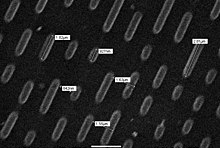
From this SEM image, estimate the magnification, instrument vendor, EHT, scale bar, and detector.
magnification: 8.5 K X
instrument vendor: JEOL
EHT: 10 kV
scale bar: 2000 nm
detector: SEI